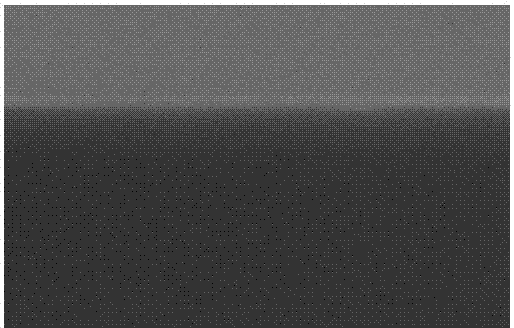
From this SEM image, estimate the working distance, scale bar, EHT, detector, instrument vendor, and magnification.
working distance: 7.3 mm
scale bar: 300 nm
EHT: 3 kV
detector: SE(M,LA3)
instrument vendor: Hitachi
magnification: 150 K X